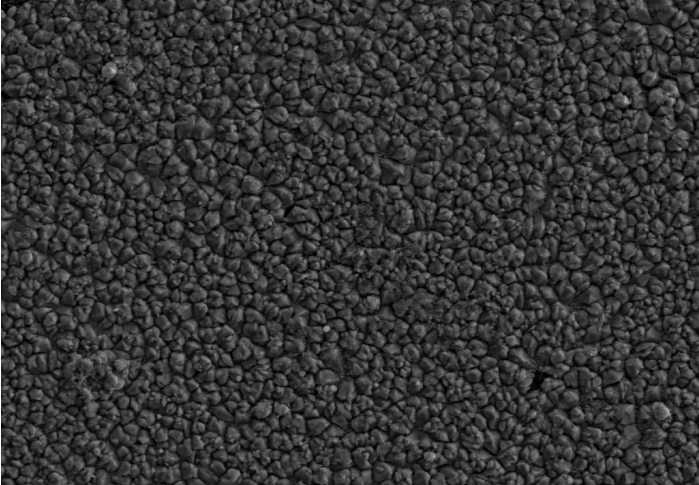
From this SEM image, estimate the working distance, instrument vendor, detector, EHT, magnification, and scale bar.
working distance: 9.4 mm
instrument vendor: Zeiss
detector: SE2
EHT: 10 kV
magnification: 1 K X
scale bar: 10000 nm